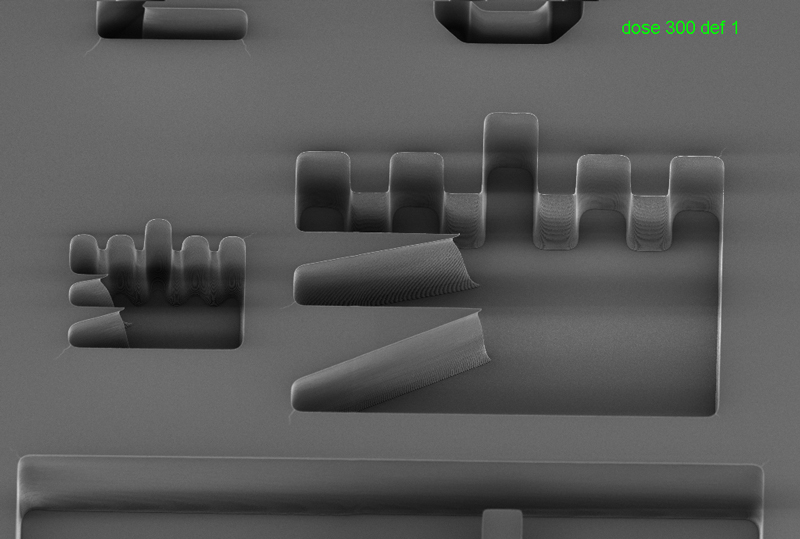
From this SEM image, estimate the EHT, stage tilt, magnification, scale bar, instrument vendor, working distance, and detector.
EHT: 1.5 kV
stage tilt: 30°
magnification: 1.36 K X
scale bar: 10000 nm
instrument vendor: Zeiss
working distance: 4.7 mm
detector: InLens